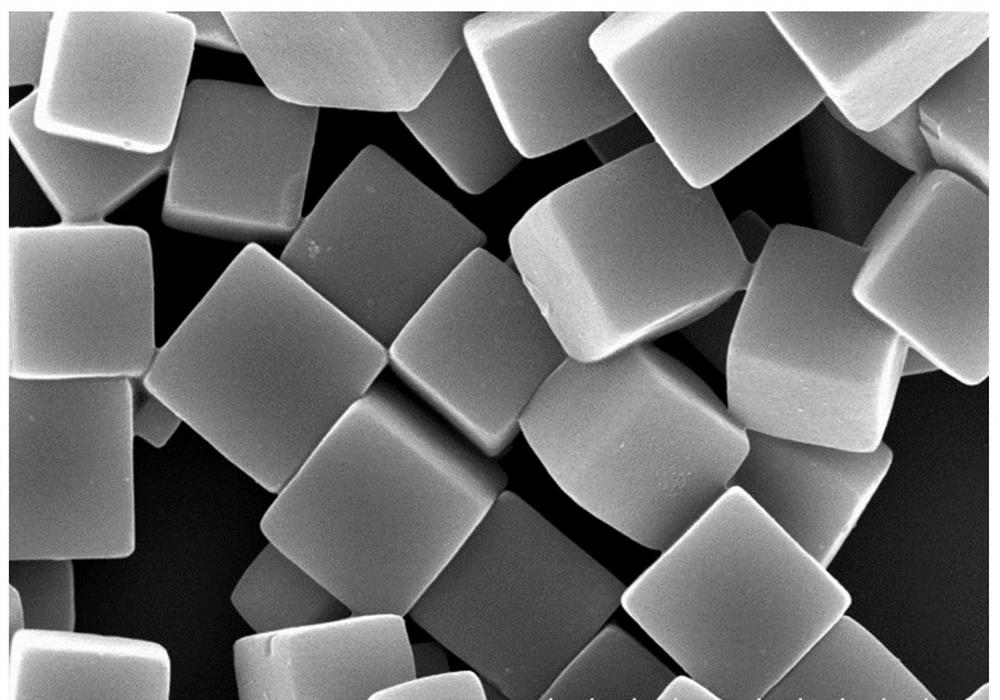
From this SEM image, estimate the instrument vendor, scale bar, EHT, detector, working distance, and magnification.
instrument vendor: Hitachi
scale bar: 3000 nm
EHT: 15 kV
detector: SE(U)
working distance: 12.7 mm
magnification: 18 K X